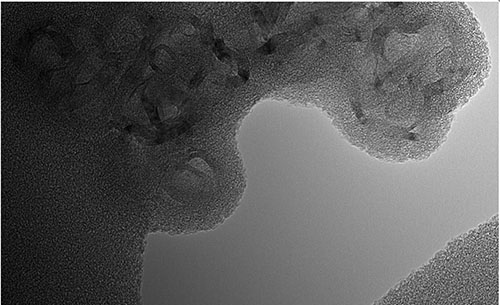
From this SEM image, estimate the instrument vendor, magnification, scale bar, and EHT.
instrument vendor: other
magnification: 250 K X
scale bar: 20 nm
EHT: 120 kV